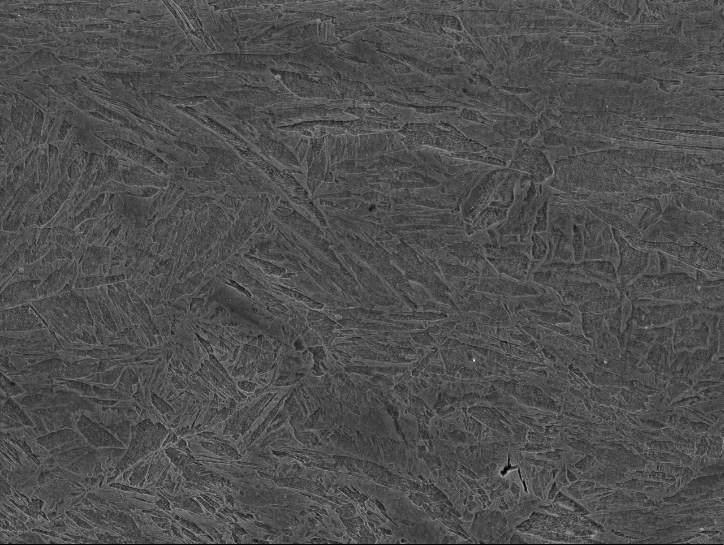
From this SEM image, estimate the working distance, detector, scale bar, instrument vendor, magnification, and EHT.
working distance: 10.3 mm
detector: SEI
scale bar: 1000 nm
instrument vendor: JEOL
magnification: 4.5 K X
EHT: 15 kV